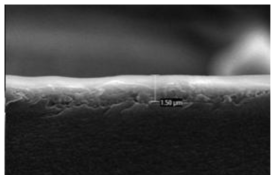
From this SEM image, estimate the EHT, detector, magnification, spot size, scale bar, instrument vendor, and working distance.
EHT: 10 kV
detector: SE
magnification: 20 K X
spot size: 3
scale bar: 2000 nm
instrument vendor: FEI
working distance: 5.1 mm